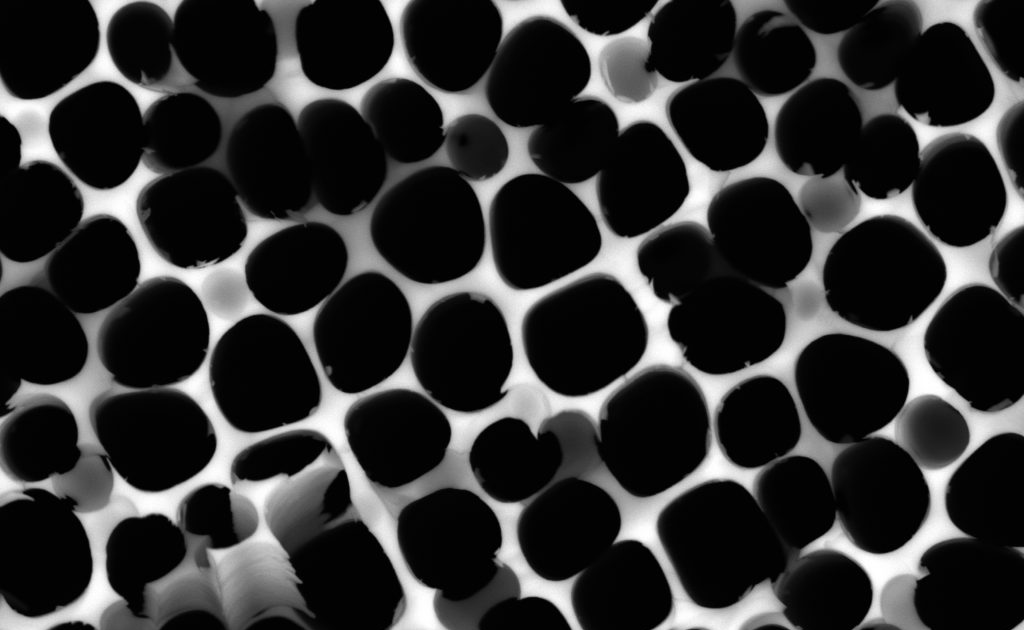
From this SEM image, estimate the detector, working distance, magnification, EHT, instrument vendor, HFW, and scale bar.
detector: BSD Full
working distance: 5.557 mm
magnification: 16 K X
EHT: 15 kV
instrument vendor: other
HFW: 39.3 µm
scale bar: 10000 nm